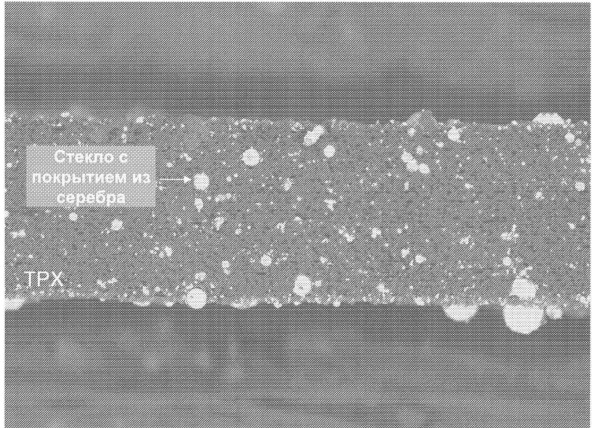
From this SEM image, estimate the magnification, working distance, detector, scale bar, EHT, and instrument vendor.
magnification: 0.2 K X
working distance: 16 mm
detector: COMPO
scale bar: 100000 nm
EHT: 10 kV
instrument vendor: JEOL